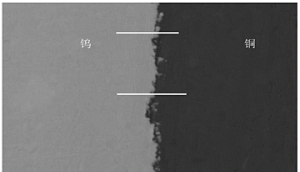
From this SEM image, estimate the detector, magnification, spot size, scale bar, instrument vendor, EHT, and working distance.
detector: BEC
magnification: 1 K X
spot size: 59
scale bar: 10000 nm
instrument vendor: JEOL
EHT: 20 kV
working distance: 15 mm